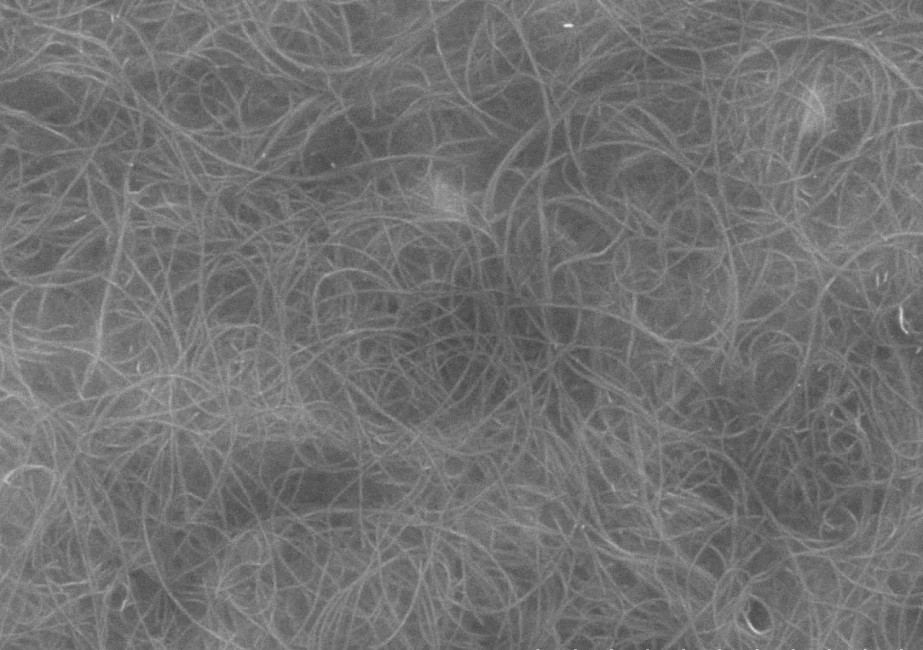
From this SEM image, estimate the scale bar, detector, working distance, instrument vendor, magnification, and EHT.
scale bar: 5000 nm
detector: SE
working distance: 5.9 mm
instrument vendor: Hitachi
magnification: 10 K X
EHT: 20 kV